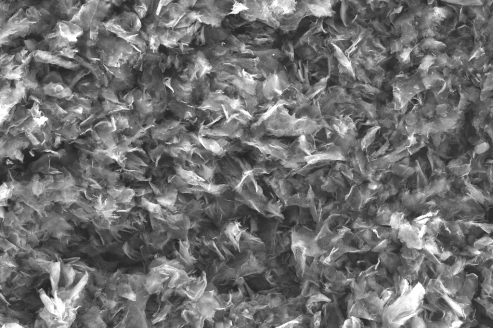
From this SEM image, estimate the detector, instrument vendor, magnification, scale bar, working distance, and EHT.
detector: SE2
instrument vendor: Zeiss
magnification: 2 K X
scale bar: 20000 nm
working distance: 10.5 mm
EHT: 20 kV